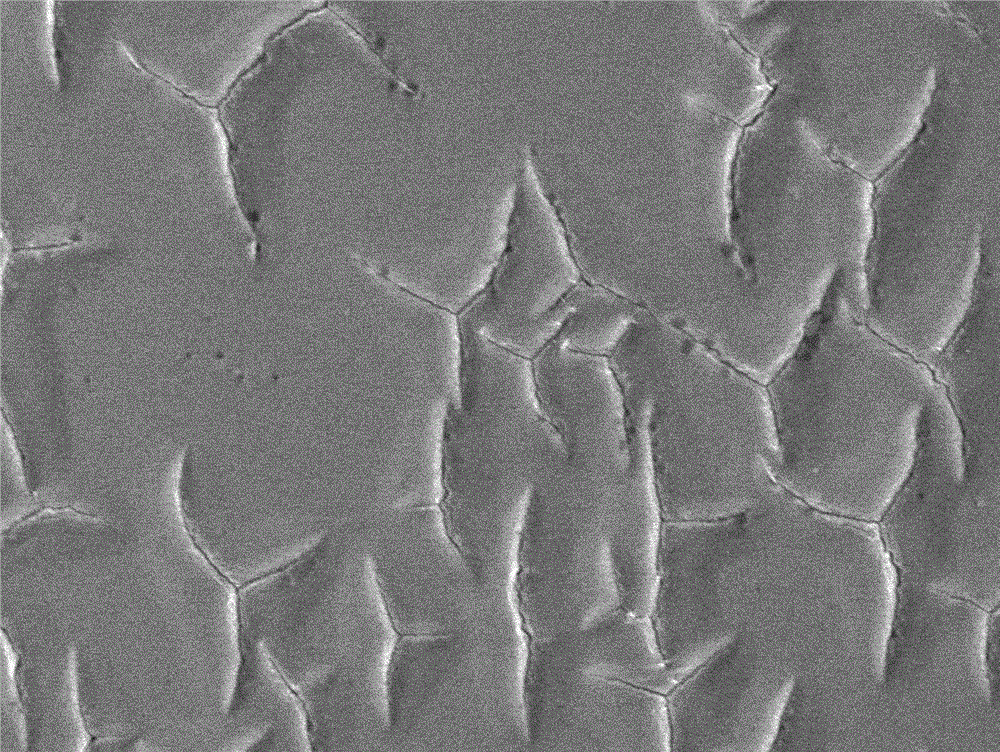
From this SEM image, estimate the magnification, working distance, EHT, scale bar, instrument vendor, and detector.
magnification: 0.2 K X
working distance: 7.9 mm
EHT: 10 kV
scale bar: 100000 nm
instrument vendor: JEOL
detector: LEI